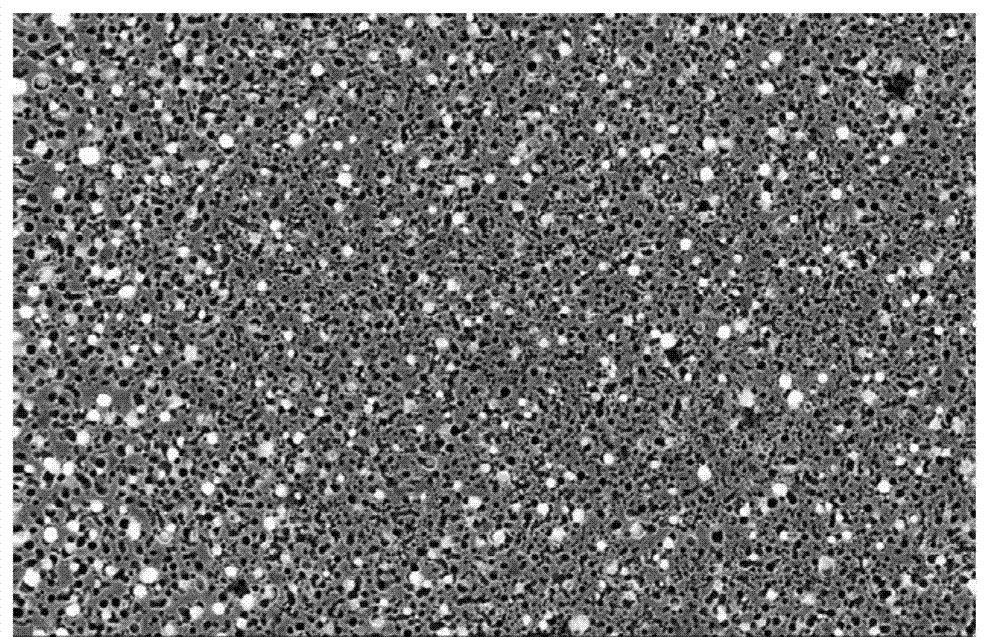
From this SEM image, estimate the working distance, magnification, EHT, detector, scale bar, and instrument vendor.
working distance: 6.7 mm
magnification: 50 K X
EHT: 5 kV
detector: InLens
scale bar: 200 nm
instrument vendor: Zeiss